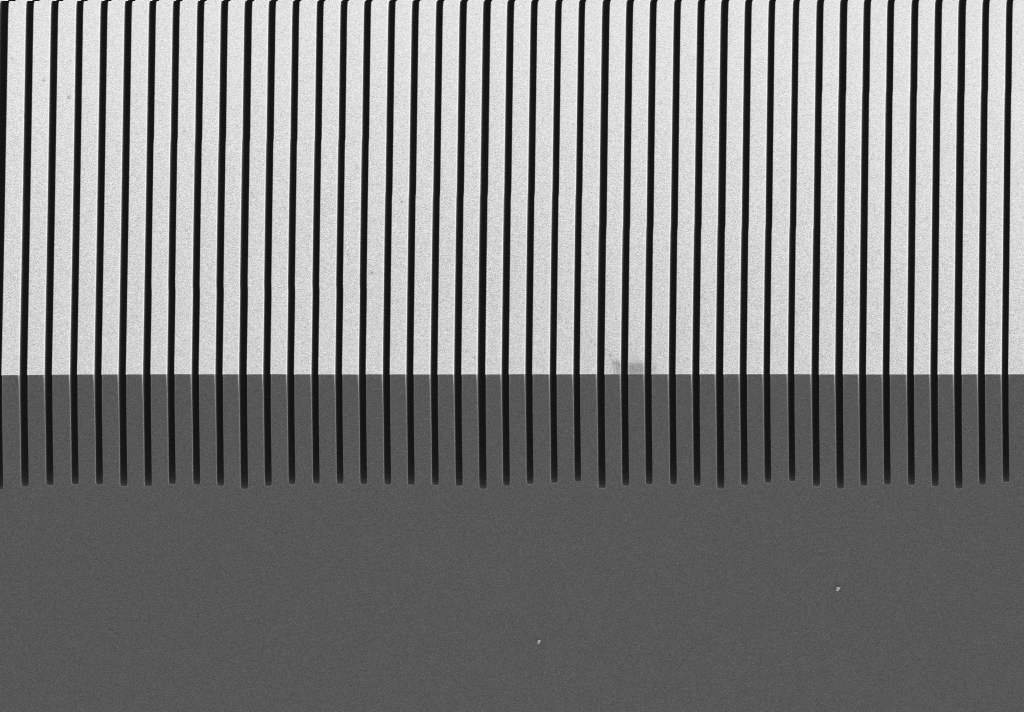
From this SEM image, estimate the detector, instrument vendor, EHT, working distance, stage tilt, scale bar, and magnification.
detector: SE2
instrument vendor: Zeiss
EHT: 5 kV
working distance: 13.7 mm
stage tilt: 45°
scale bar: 10000 nm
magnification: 0.5 K X